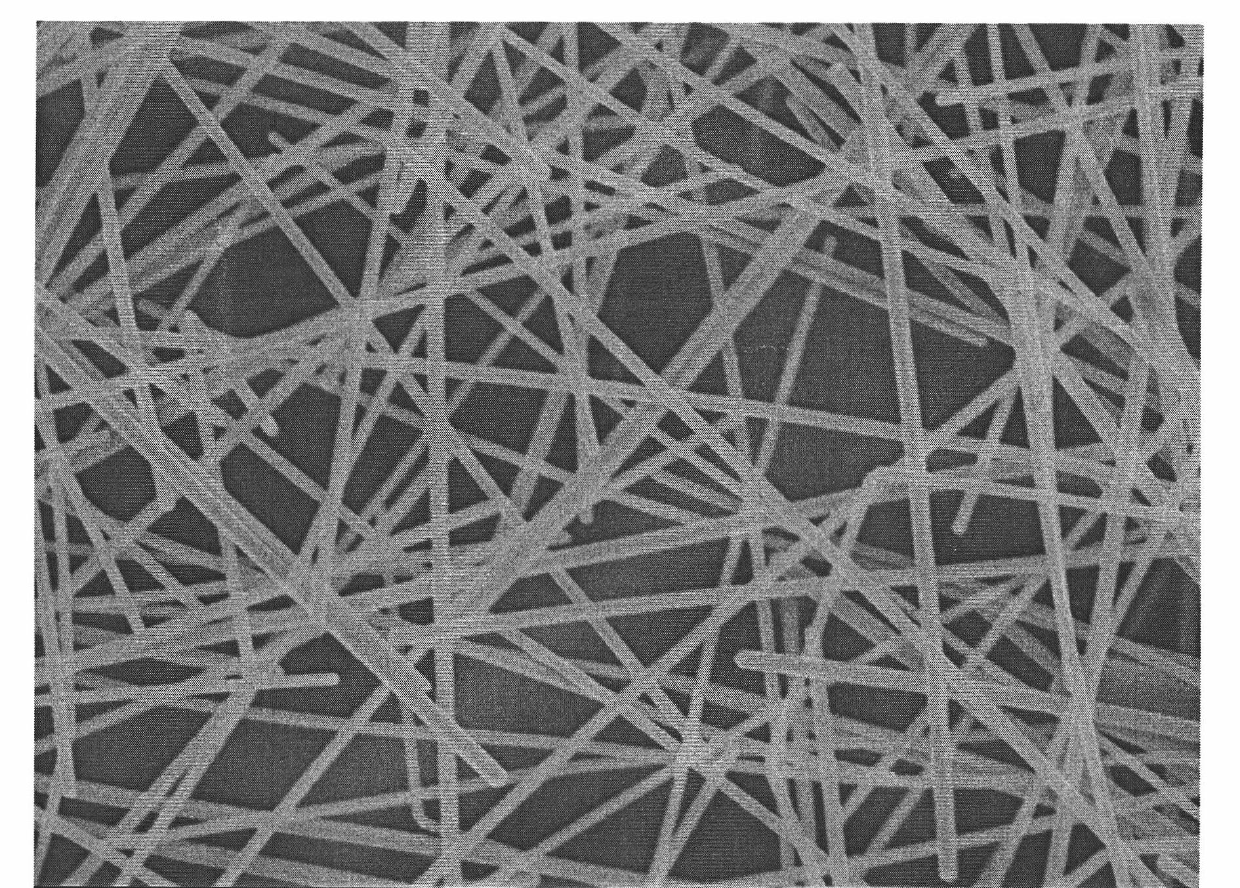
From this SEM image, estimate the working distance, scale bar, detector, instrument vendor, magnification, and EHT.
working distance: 8.1 mm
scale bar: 100 nm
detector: SEI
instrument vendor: JEOL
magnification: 30 K X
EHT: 5 kV